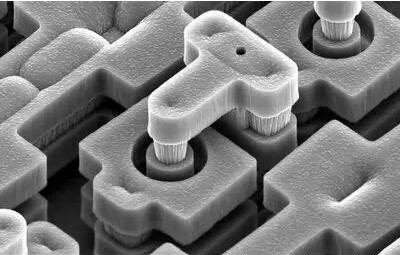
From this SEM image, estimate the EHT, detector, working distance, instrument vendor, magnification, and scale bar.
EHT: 10 kV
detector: SE(M)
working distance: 12.5 mm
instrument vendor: Hitachi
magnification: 5 K X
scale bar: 10000 nm